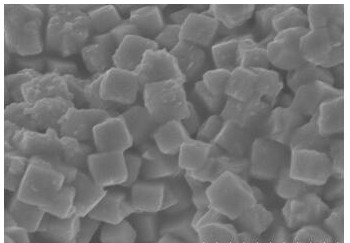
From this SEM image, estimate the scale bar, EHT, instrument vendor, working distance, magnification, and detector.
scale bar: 1000 nm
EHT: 5 kV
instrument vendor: Hitachi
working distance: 4.9 mm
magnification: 50 K X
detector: SE(M)